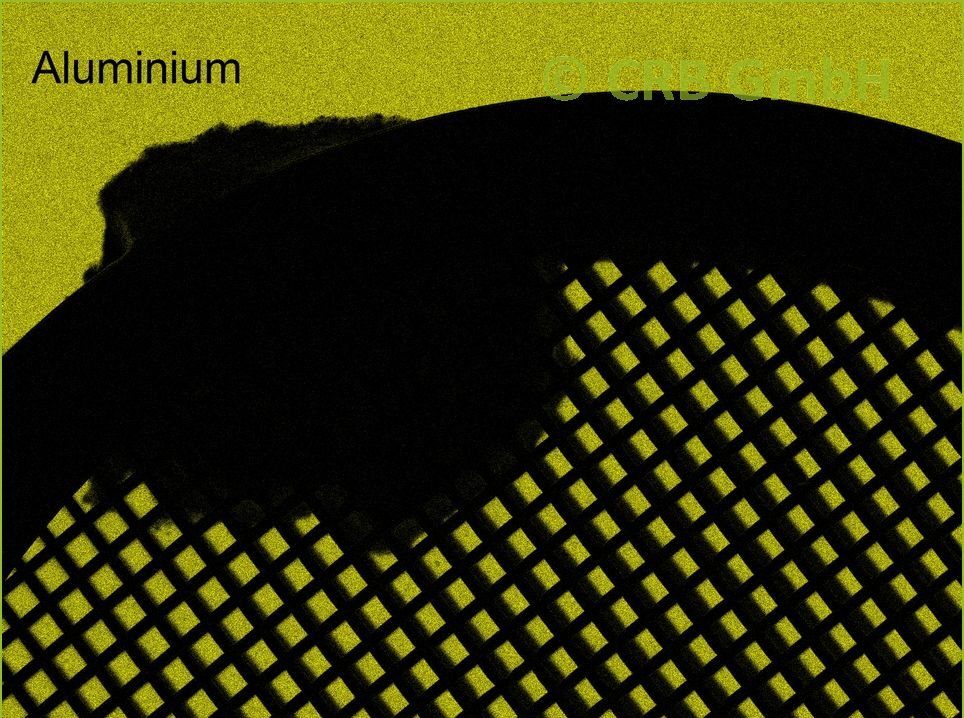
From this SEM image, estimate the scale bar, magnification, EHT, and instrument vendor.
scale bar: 200000 nm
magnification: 0.062 K X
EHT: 20 kV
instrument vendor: other